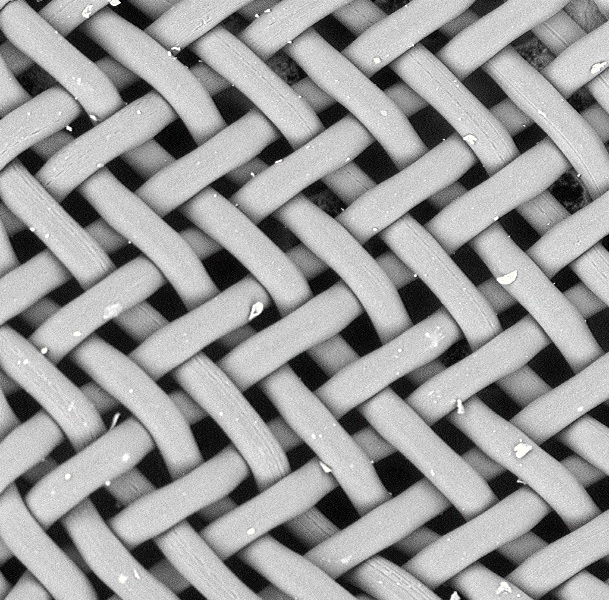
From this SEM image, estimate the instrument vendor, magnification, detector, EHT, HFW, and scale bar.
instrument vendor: other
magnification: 0.35 K X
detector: BSD Full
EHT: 15 kV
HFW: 778 µm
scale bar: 200000 nm